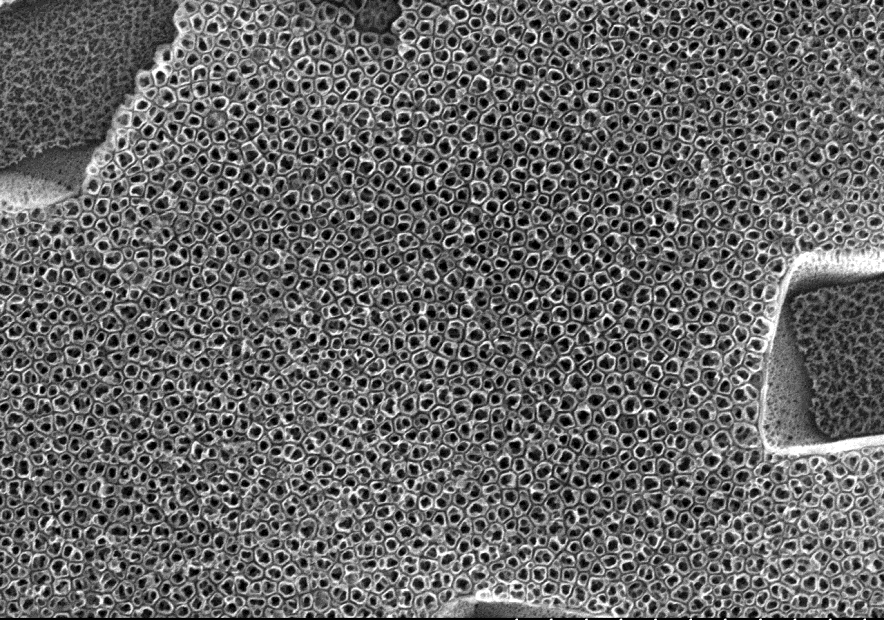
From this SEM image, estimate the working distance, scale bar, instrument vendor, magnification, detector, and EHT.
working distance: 12.6 mm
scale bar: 2000 nm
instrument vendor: Hitachi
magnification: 25 K X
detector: SE(M)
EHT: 15 kV